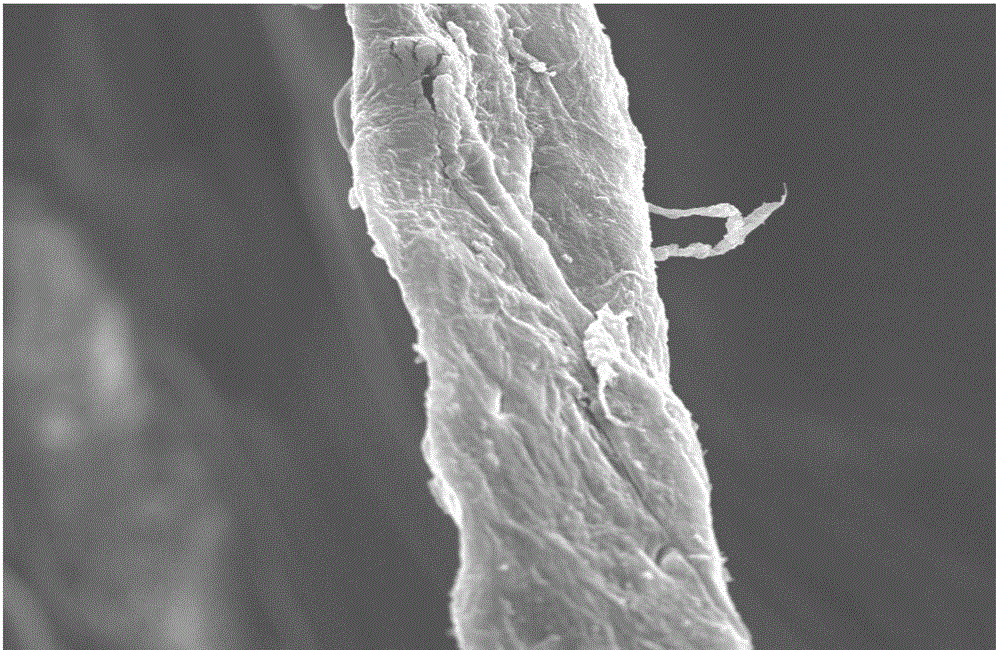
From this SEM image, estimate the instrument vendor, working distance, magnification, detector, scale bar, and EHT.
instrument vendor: JEOL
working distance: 8.6 mm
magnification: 2 K X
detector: SEI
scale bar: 10000 nm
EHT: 15 kV